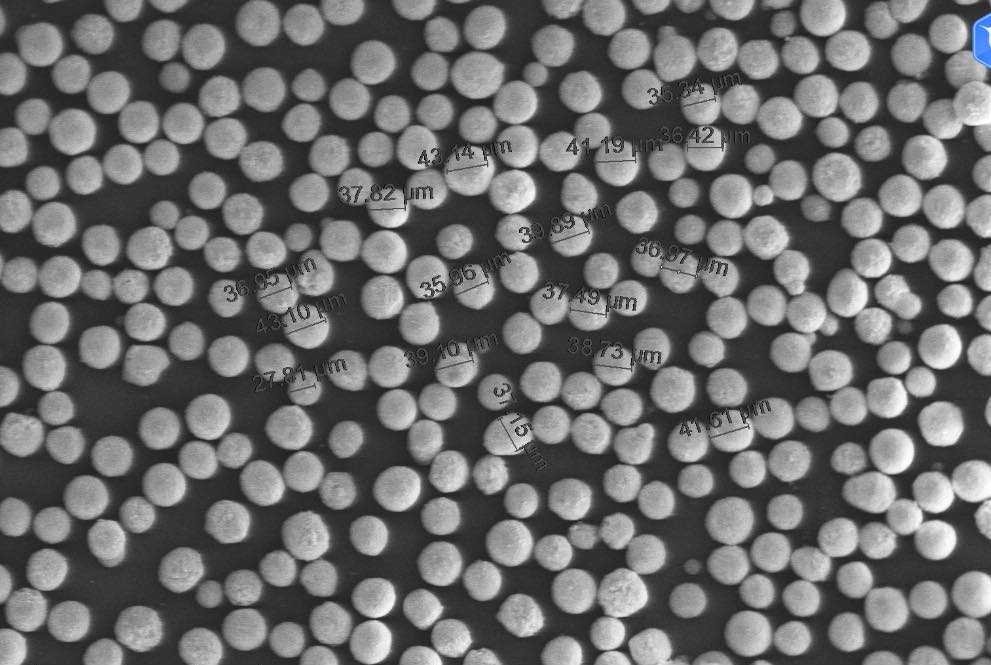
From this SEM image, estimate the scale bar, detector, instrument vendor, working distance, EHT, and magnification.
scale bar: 400000 nm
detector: ETD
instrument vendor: FEI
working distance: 5.6 mm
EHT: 15 kV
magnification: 0.4 K X